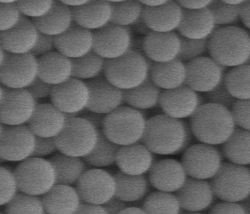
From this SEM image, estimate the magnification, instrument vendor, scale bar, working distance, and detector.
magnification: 20 K X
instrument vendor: FEI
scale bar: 1000 nm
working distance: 4.9 mm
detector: SE